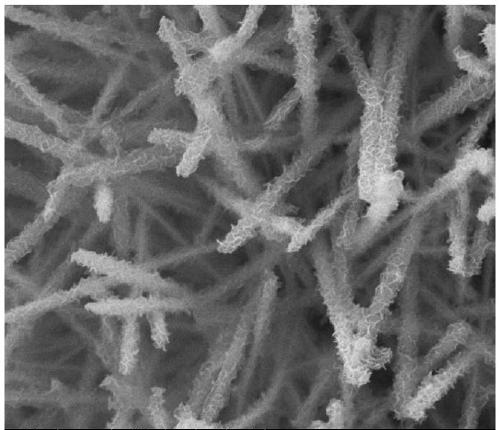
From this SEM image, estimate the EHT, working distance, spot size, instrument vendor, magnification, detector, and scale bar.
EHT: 30 kV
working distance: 10.4 mm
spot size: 3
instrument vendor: FEI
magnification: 20 K X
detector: ETD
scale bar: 5000 nm